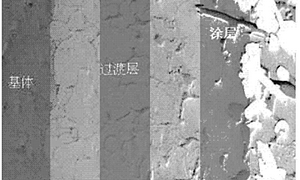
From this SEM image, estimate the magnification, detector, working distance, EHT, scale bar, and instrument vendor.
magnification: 0.25 K X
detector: SE(L)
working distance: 12.1 mm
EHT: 15 kV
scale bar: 200000 nm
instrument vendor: Hitachi